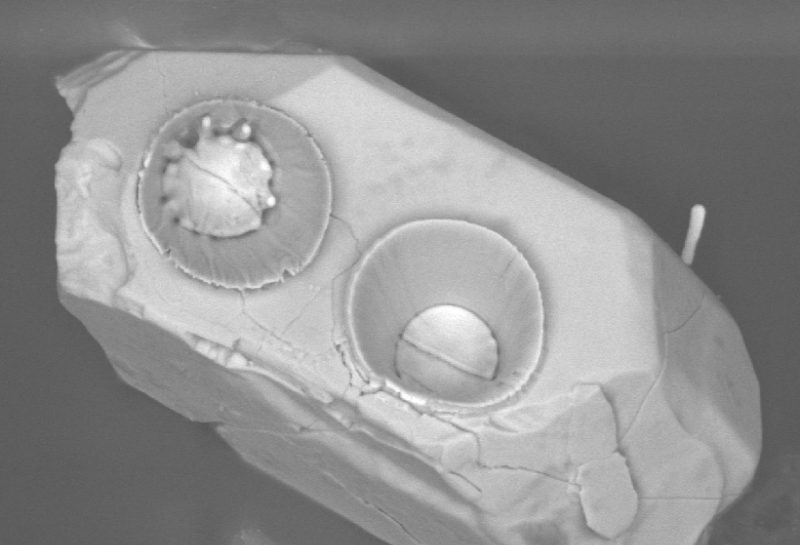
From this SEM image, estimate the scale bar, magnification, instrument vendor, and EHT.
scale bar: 10000 nm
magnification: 1 K X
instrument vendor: JEOL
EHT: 20 kV